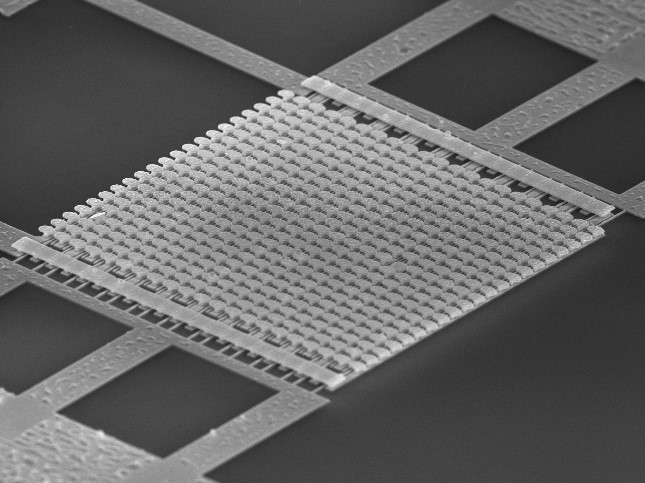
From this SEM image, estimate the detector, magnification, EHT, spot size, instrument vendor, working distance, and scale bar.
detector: TLD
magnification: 4.231 K X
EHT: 15 kV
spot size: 3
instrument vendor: FEI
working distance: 4.8 mm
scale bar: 5000 nm